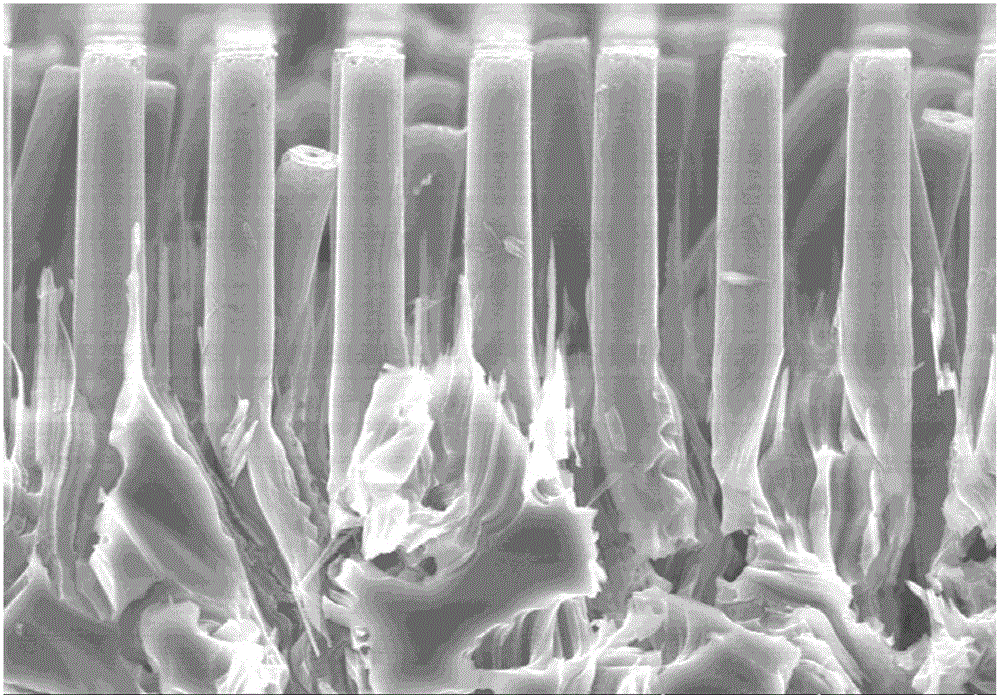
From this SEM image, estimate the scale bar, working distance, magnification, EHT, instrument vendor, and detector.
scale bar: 20000 nm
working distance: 9.4 mm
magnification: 2 K X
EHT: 15 kV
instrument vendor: Hitachi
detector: SE(UL)